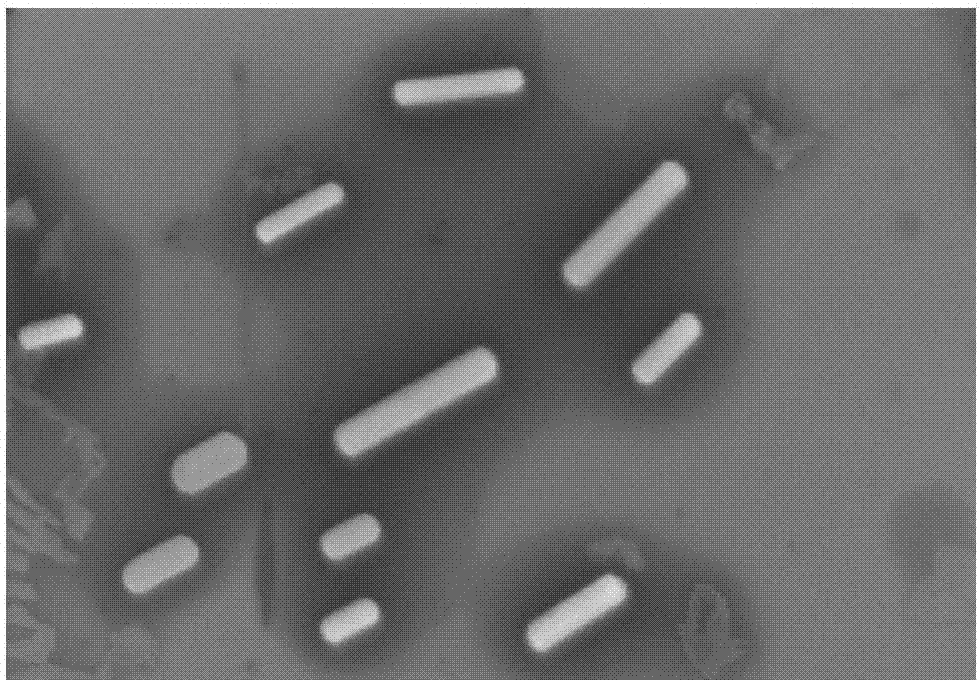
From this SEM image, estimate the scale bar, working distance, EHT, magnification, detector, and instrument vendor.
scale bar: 2000 nm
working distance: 9 mm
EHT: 15 kV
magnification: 20 K X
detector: SE(M)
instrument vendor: Hitachi